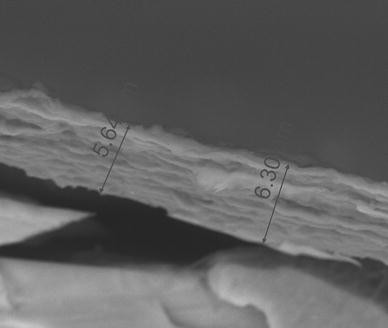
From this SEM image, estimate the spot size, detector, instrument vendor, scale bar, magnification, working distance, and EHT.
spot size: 3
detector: GAD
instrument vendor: FEI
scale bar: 5000 nm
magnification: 5 K X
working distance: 11.1 mm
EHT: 15 kV